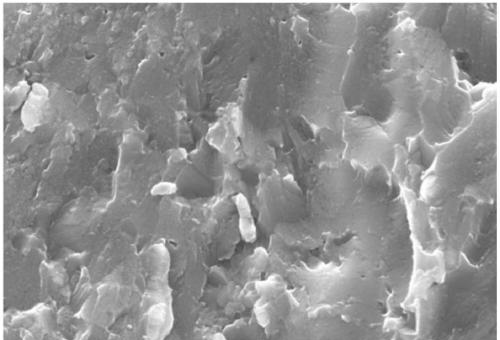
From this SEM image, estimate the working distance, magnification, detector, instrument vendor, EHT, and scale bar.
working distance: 9 mm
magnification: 10 K X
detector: SE1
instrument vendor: Zeiss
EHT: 20 kV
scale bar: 1000 nm